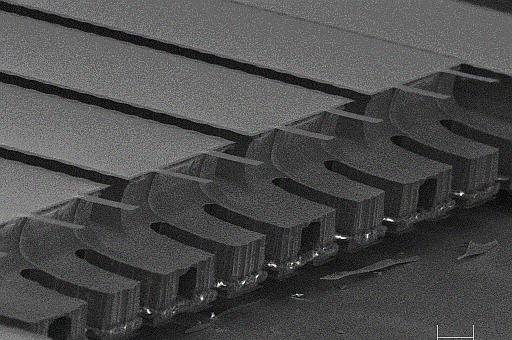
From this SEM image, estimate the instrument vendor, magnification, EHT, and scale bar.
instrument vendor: JEOL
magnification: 0.04 K X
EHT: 25 kV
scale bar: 200000 nm